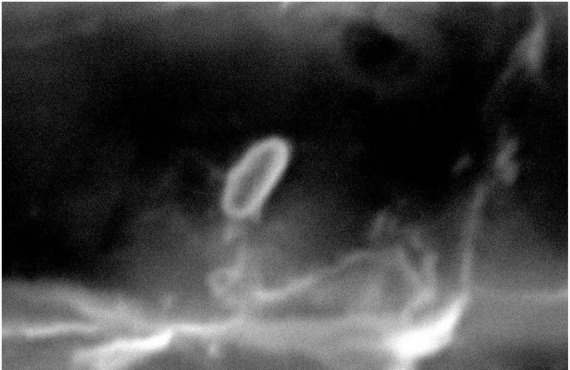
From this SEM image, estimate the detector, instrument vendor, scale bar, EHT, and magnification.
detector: SEI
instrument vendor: JEOL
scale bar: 1000 nm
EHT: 20 kV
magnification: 15 K X